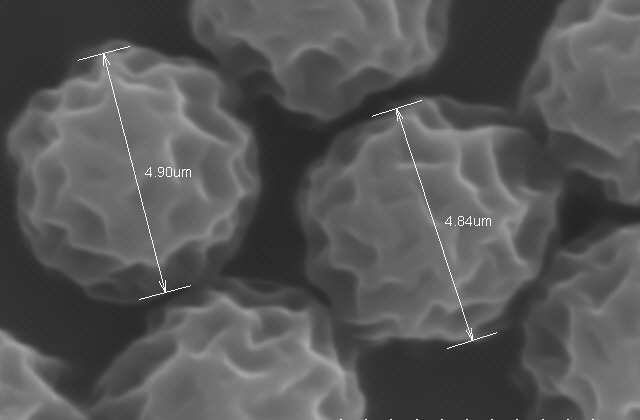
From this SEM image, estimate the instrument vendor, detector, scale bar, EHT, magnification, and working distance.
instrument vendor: Hitachi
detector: SE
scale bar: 5000 nm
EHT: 15 kV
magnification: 10 K X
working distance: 10.3 mm